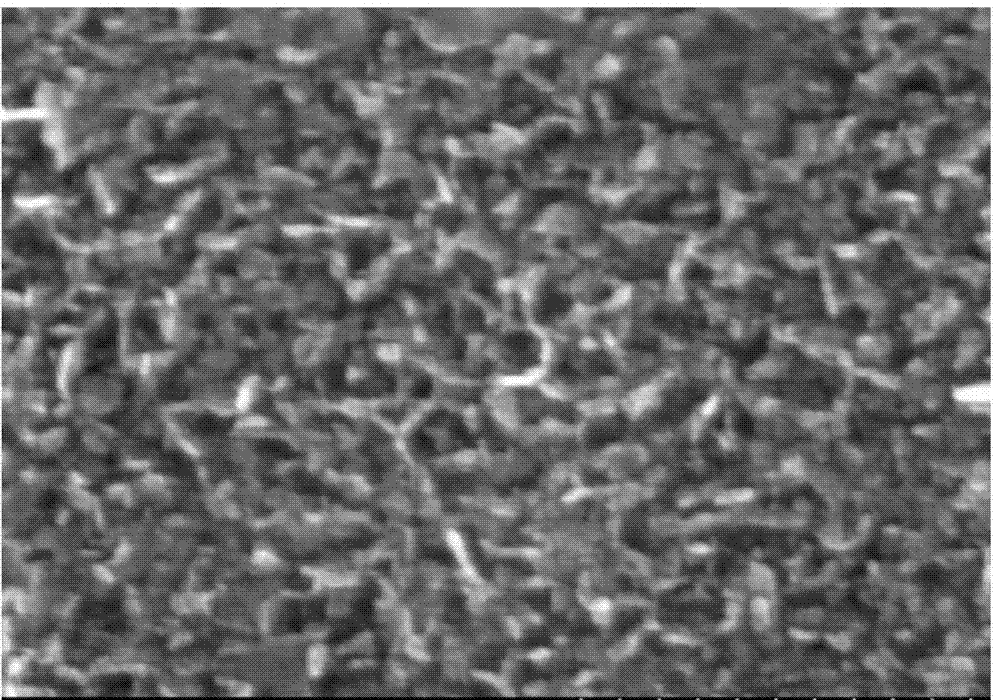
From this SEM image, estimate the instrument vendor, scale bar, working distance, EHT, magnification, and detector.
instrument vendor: Hitachi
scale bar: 500 nm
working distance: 4.6 mm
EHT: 5 kV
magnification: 100 K X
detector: SE(M)